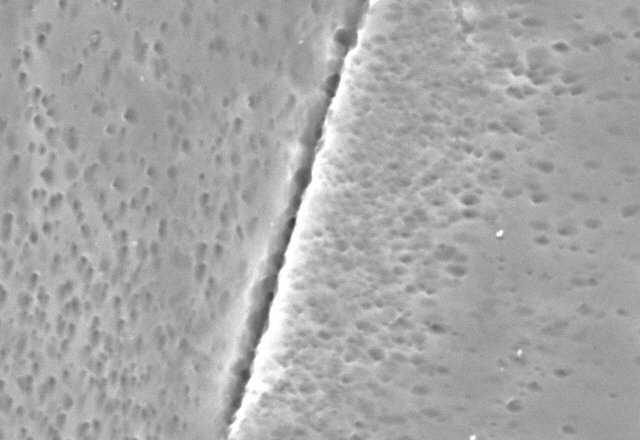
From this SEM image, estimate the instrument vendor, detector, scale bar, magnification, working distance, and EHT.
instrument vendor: other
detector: SE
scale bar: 10000 nm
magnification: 5 K X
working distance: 10.1 mm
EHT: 25 kV